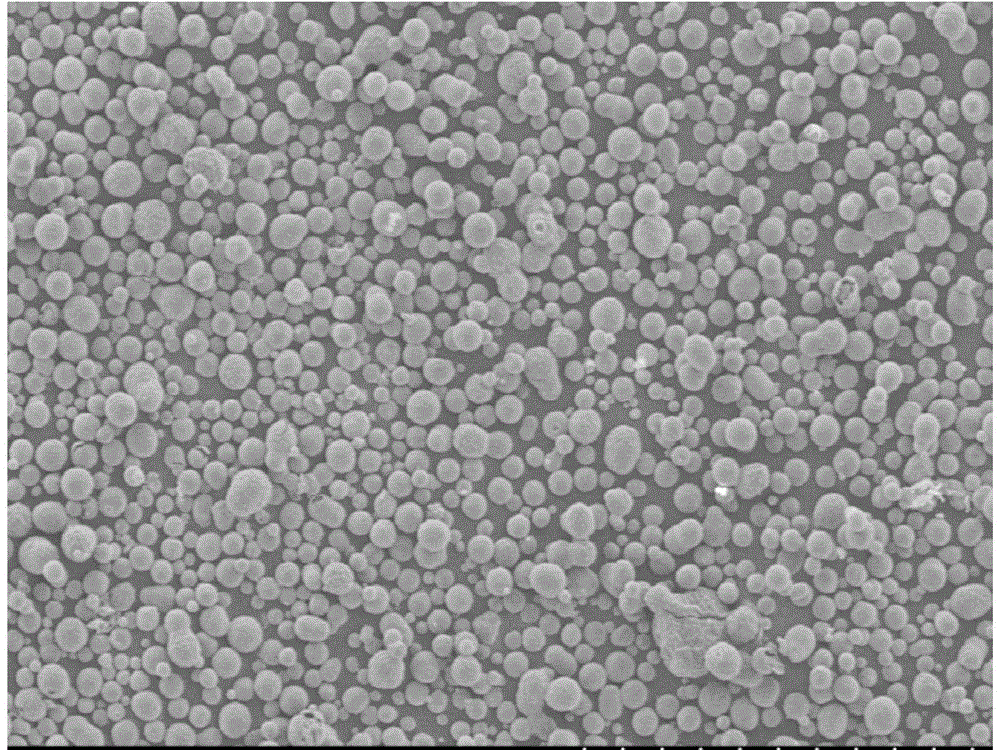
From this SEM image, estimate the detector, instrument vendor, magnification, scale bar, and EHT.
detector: SE(M)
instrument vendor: Hitachi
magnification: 0.1 K X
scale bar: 500000 nm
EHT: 5 kV